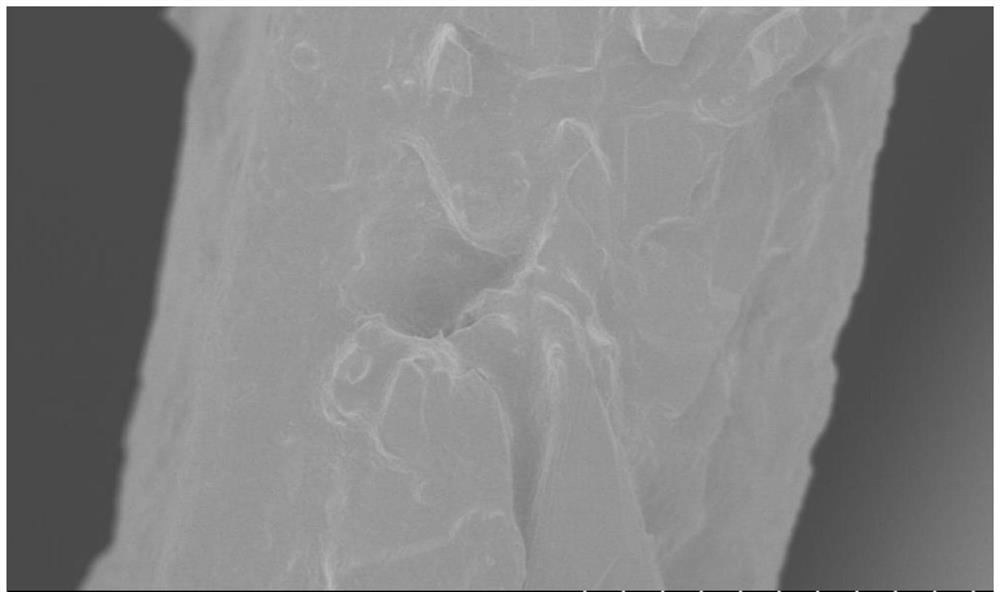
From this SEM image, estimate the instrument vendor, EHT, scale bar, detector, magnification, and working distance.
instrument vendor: Hitachi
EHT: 1 kV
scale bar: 5000 nm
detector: SE(U)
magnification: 10 K X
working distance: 4.2 mm